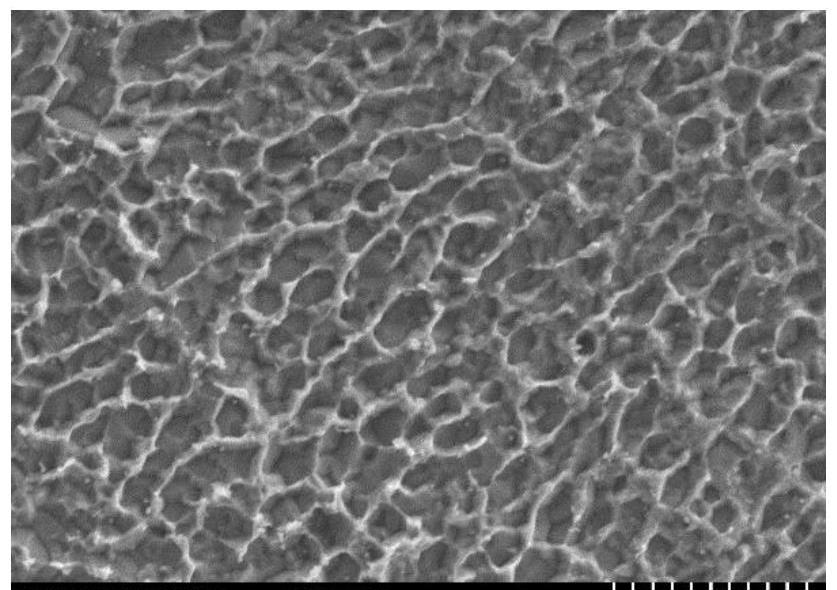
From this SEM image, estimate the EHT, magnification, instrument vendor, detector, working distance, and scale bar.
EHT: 15 kV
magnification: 3 K X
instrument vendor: Hitachi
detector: SE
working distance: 7.5 mm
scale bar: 10000 nm